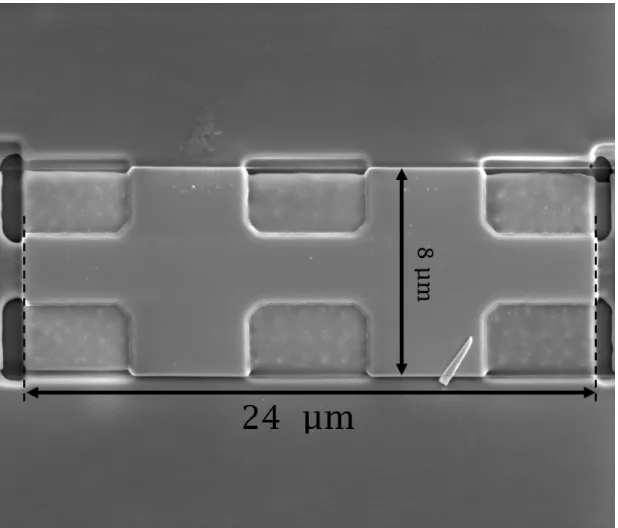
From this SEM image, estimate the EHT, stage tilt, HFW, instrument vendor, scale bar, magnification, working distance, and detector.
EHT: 5 kV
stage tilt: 0°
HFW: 25.6 µm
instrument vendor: FEI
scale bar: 5000 nm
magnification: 10 K X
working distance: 5.1 mm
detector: SE